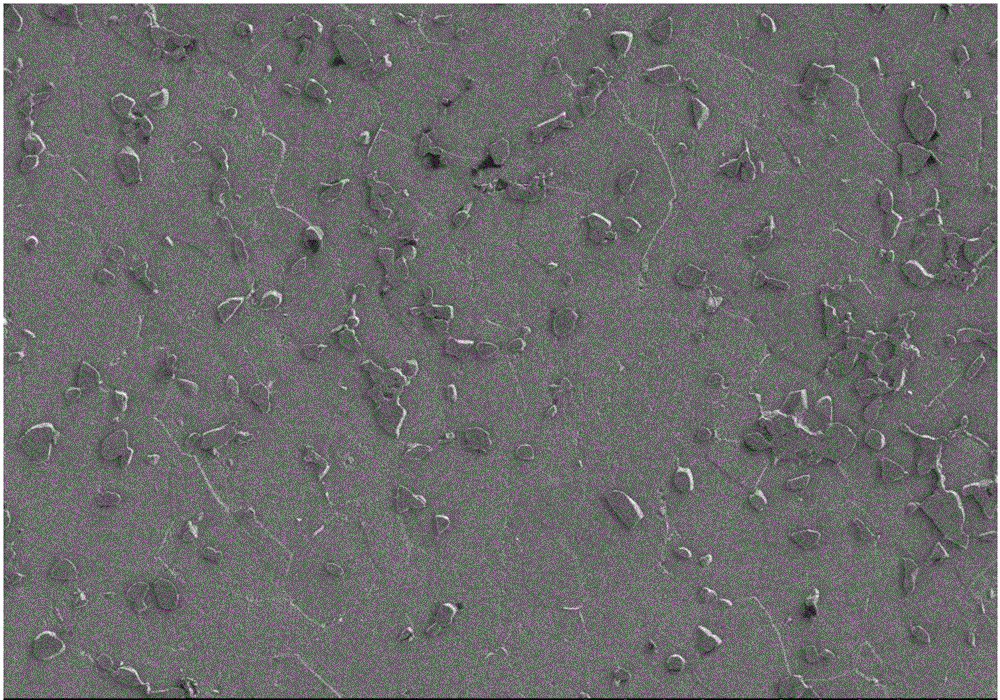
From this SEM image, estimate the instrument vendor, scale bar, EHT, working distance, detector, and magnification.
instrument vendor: Hitachi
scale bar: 100000 nm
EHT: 5 kV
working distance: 8.1 mm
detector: SE(M)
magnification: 0.5 K X